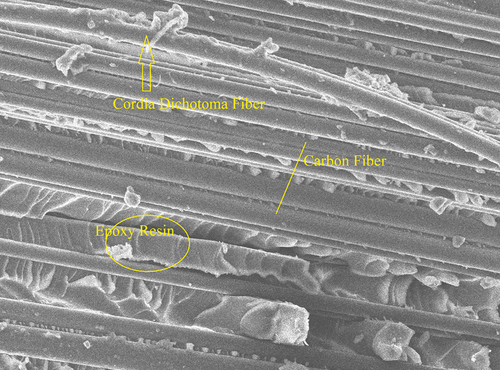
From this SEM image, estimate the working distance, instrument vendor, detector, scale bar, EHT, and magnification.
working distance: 14.4 mm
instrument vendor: JEOL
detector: SED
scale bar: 20000 nm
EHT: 10 kV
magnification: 0.85 K X